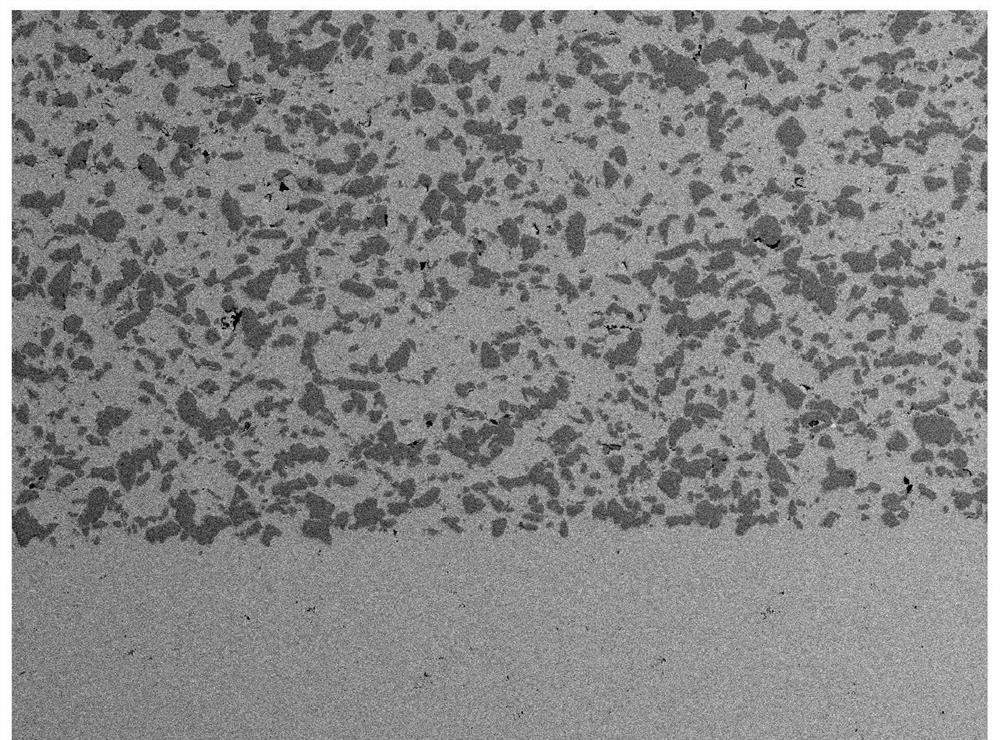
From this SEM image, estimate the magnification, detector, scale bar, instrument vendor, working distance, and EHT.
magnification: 0.1 K X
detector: BSE2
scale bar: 500000 nm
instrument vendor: JEOL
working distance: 24.2 mm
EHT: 23 kV